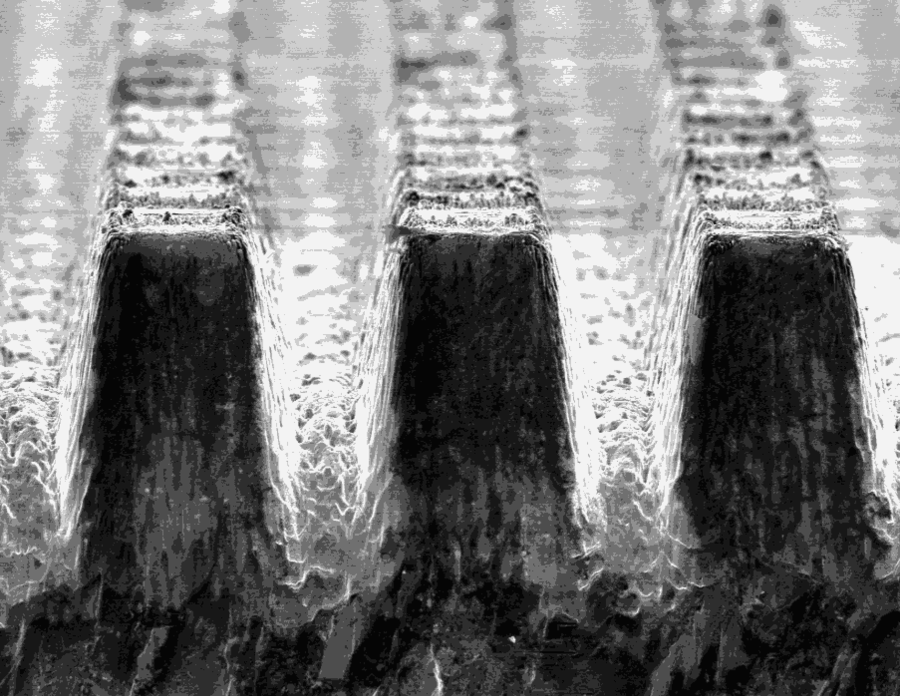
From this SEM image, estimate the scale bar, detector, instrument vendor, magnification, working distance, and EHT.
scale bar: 300000 nm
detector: ETD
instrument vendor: FEI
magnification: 0.2 K X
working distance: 10.7 mm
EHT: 20 kV